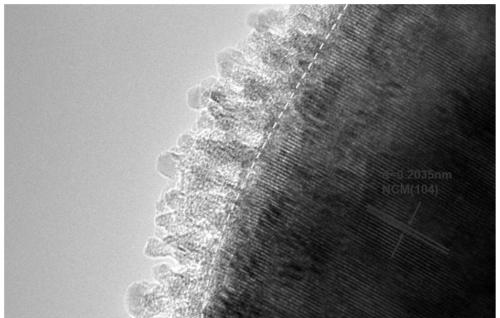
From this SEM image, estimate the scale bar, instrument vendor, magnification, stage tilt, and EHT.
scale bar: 10 nm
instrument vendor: JEOL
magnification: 800 K X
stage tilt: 0.1°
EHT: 200 kV